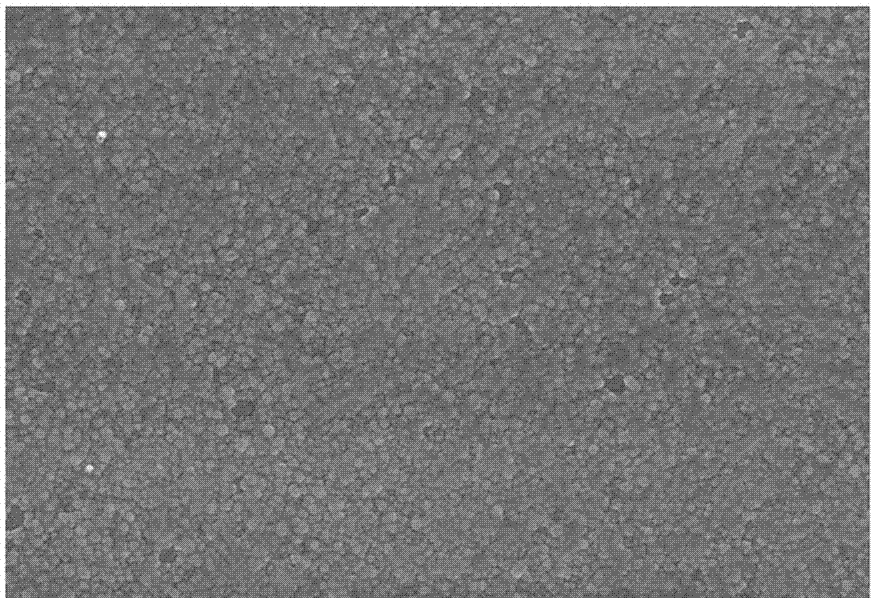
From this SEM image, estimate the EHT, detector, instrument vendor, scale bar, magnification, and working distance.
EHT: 10 kV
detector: SE(U)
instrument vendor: Hitachi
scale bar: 1000 nm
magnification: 30 K X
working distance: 8.6 mm